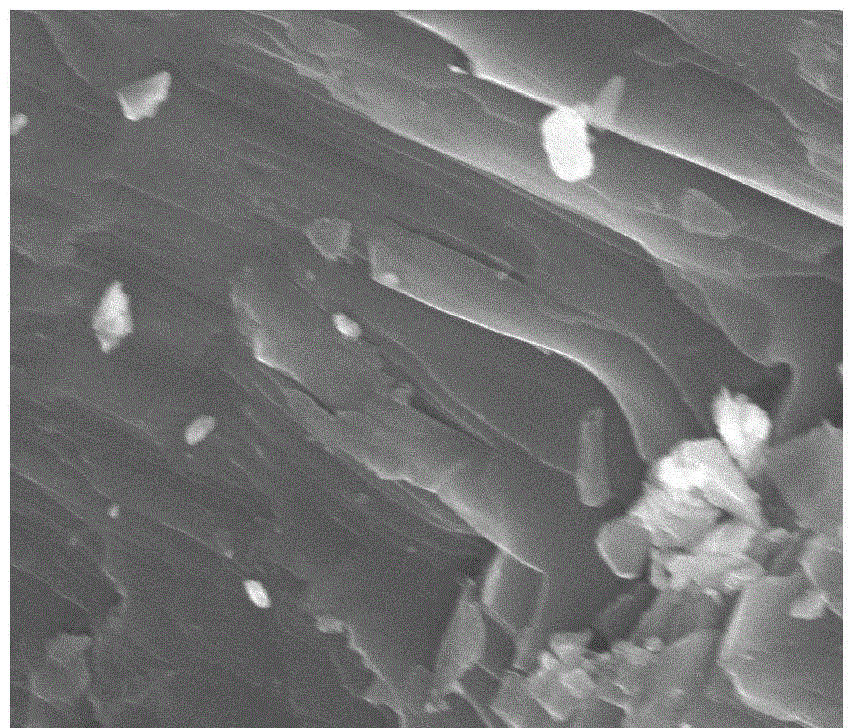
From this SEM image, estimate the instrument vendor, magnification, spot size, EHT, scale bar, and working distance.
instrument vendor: FEI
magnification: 80 K X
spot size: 2.5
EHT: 10 kV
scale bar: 1000 nm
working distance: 9.9 mm